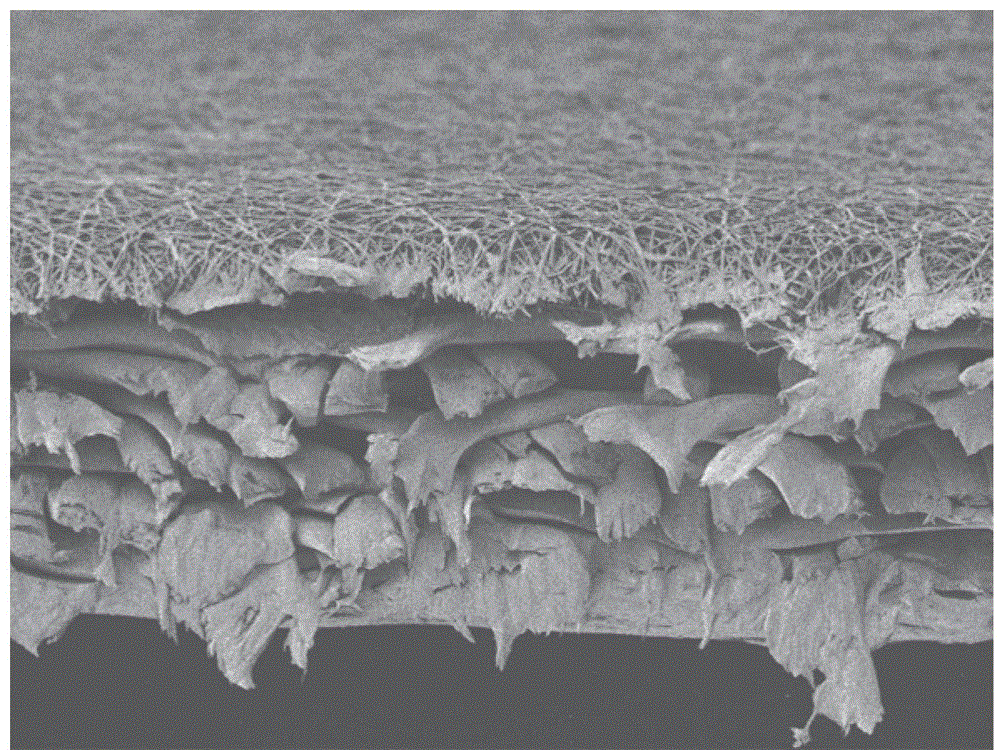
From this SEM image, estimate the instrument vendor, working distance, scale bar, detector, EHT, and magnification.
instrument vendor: JEOL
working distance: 8.1 mm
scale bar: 10000 nm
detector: LEI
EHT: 1 kV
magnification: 0.5 K X